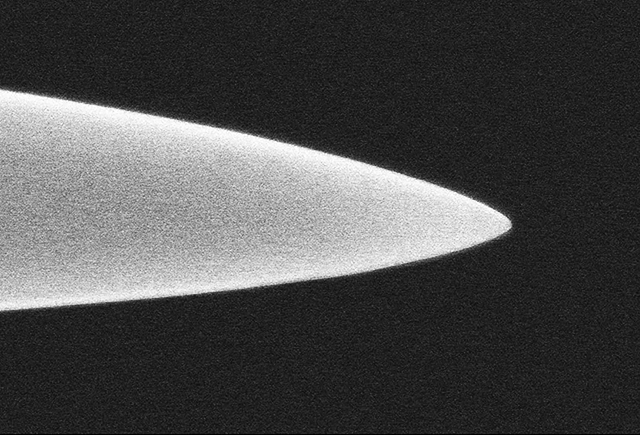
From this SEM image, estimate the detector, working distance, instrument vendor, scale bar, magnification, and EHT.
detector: SE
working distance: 18.5 mm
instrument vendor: Hitachi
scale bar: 500 nm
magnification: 100 K X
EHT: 10 kV